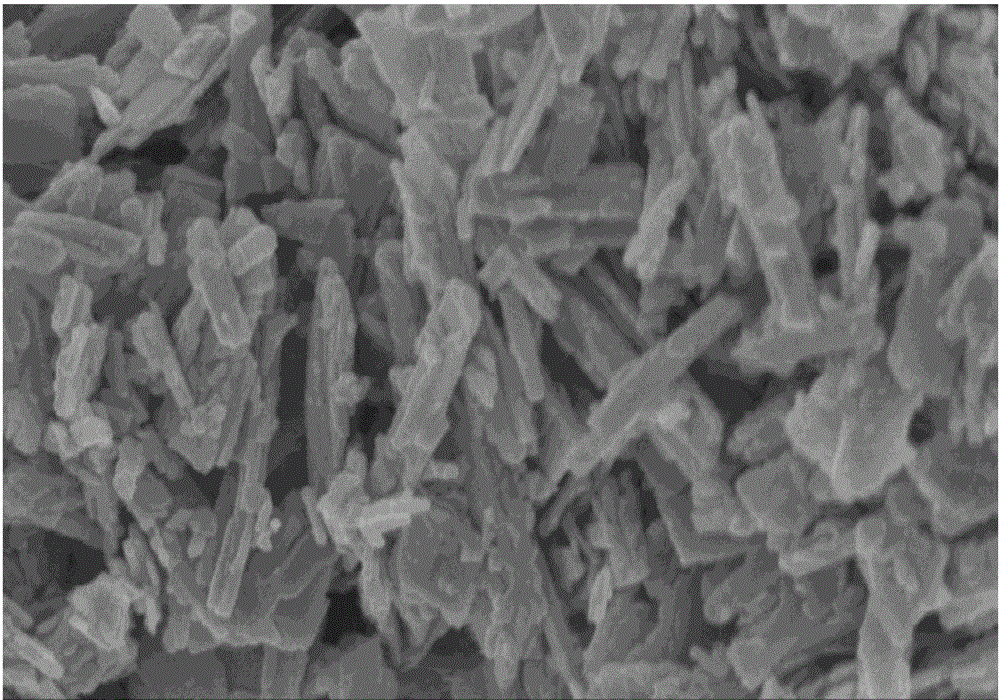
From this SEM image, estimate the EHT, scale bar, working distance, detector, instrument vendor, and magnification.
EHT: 3 kV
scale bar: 1000 nm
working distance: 9.1 mm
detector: SE(M)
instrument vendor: Hitachi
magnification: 50 K X